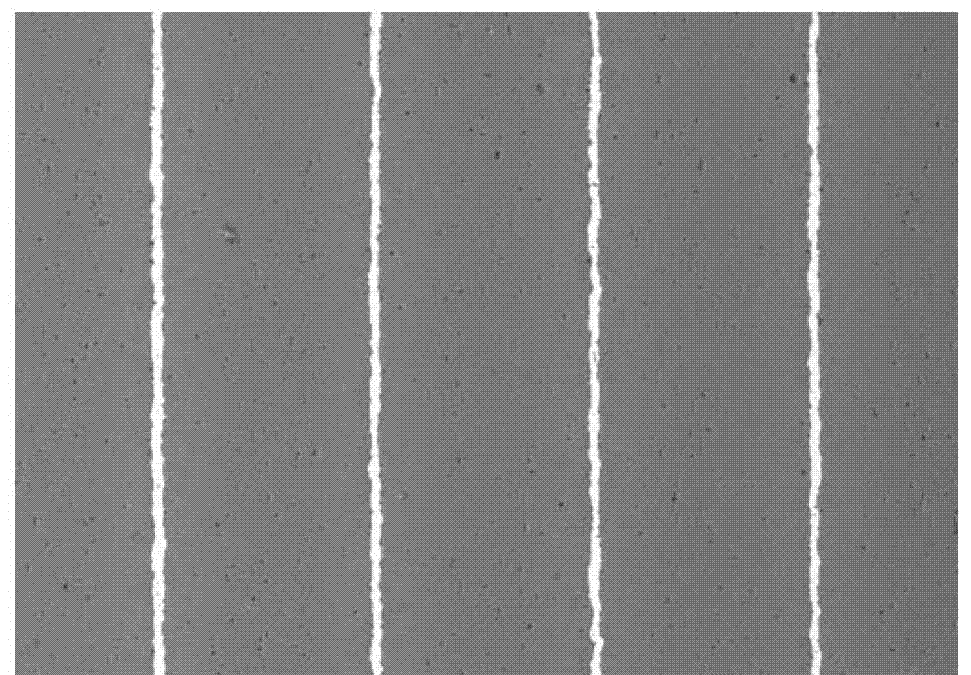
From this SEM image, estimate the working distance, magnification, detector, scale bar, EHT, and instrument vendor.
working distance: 11 mm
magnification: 0.15 K X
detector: COMP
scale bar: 100000 nm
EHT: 20 kV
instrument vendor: JEOL